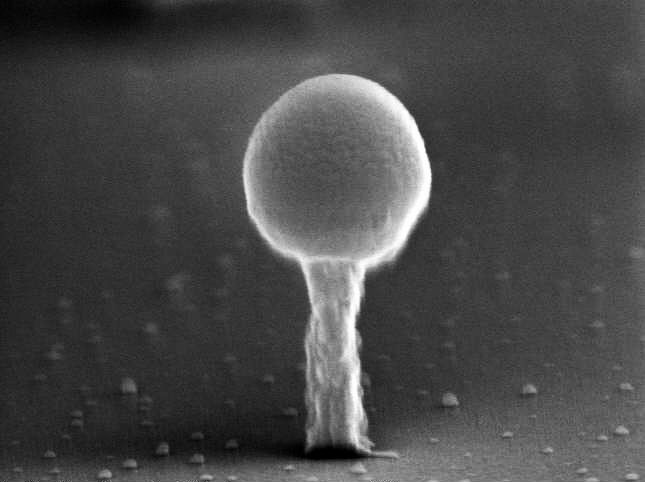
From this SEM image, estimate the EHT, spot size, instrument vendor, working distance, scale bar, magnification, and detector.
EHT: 20 kV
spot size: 3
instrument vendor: FEI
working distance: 4.7 mm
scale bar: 200 nm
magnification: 134.868 K X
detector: TLD